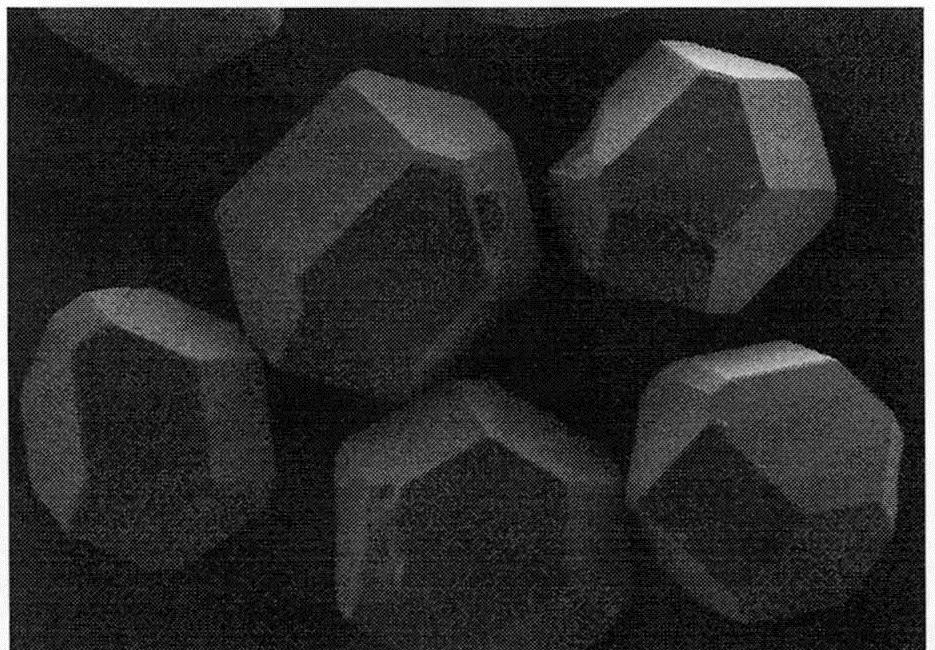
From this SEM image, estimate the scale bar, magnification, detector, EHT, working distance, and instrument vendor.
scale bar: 200000 nm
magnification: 0.2 K X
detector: SE(M)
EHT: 5 kV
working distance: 8.2 mm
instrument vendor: Hitachi